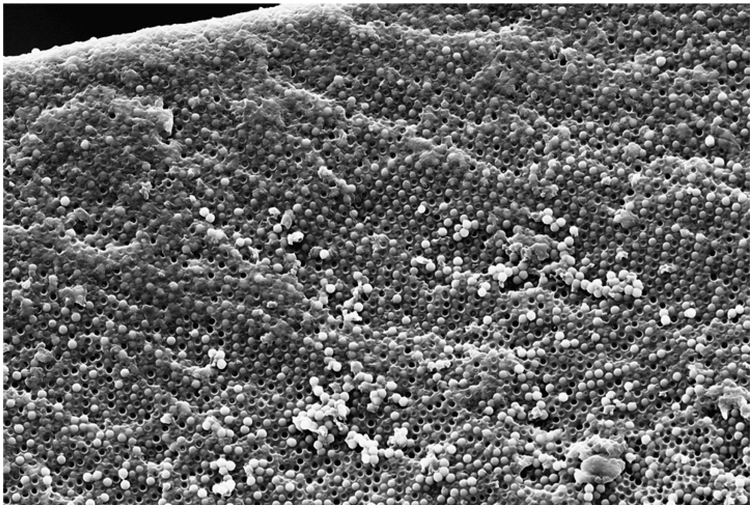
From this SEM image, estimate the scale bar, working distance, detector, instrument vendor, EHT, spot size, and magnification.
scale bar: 10000 nm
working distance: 15.2 mm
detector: SE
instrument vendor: FEI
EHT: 10 kV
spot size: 3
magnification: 2.5 K X